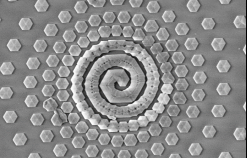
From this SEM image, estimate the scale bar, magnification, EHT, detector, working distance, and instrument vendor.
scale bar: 3000 nm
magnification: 5.08 K X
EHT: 5 kV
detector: InLens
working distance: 4.2 mm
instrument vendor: Zeiss